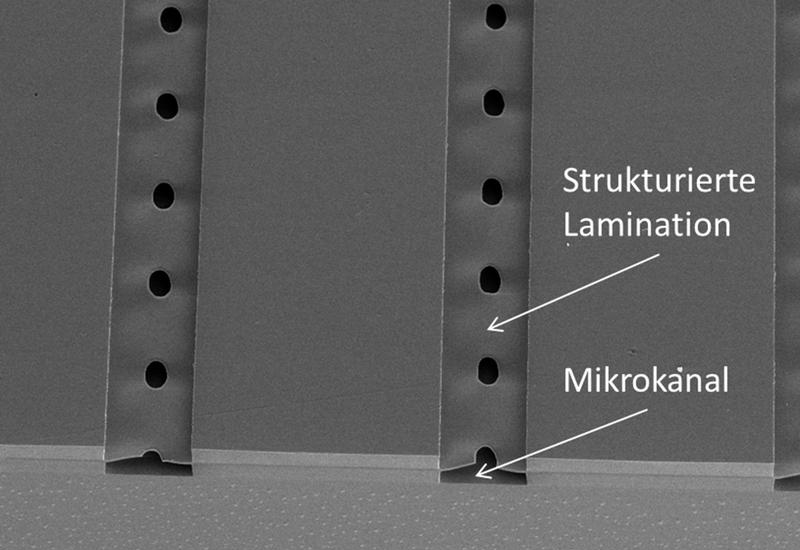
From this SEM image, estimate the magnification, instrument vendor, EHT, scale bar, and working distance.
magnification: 0.05 K X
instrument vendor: JEOL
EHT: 15 kV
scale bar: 500000 nm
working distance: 31 mm